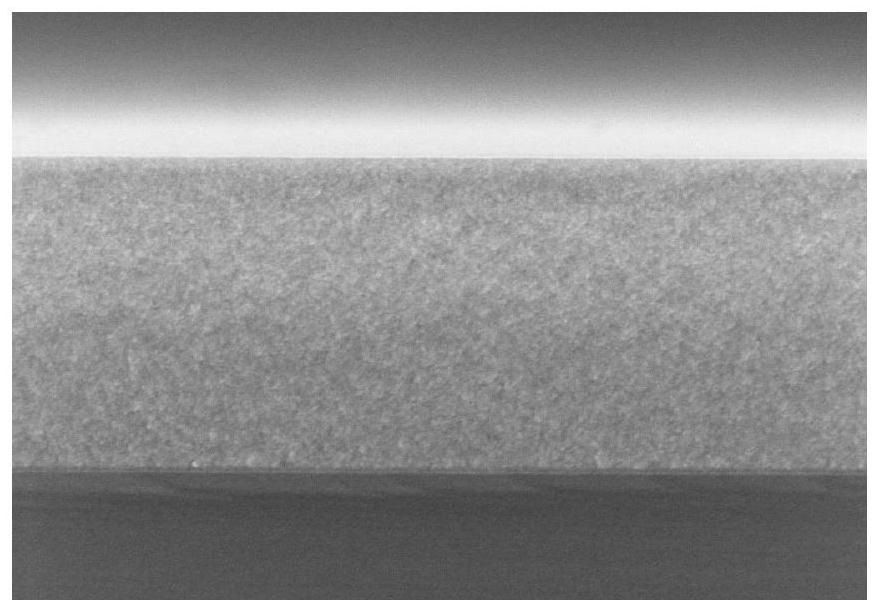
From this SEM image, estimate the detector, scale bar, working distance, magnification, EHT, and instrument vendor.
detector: InLens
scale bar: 200 nm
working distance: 3.2 mm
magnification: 50 K X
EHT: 3 kV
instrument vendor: Zeiss